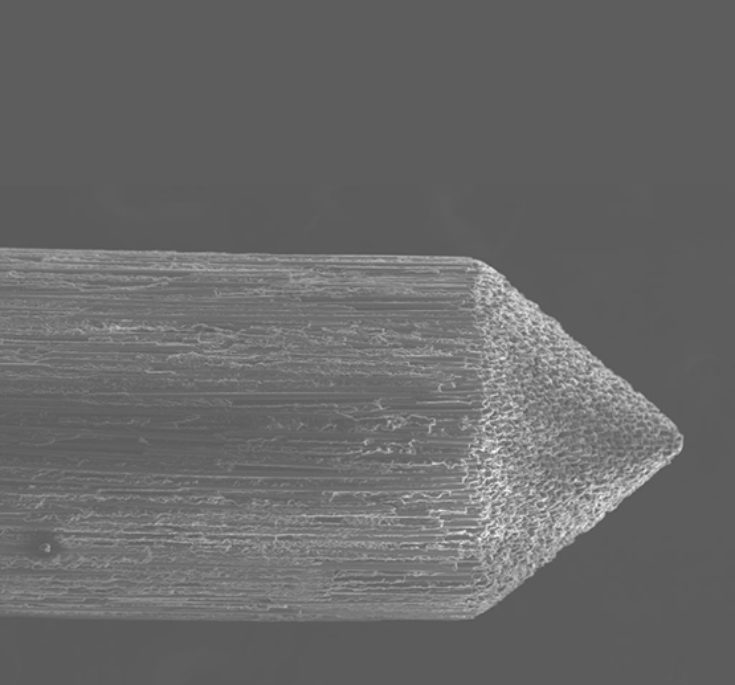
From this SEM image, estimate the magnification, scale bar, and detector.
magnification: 0.06 K X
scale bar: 400000 nm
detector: SE